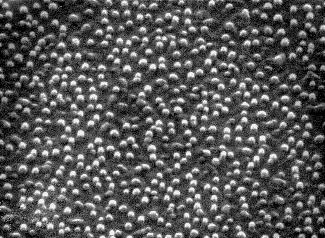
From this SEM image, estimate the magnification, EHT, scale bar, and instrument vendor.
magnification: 50 K X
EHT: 25 kV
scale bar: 600 nm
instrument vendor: JEOL